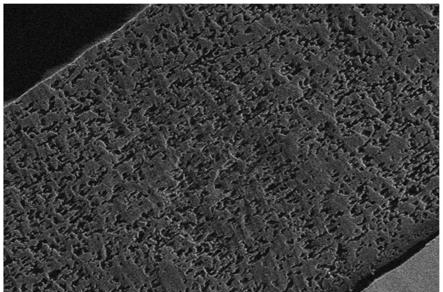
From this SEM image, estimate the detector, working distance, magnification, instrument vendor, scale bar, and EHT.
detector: SE2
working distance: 4 mm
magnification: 10 K X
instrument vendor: Zeiss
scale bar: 1000 nm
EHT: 1 kV